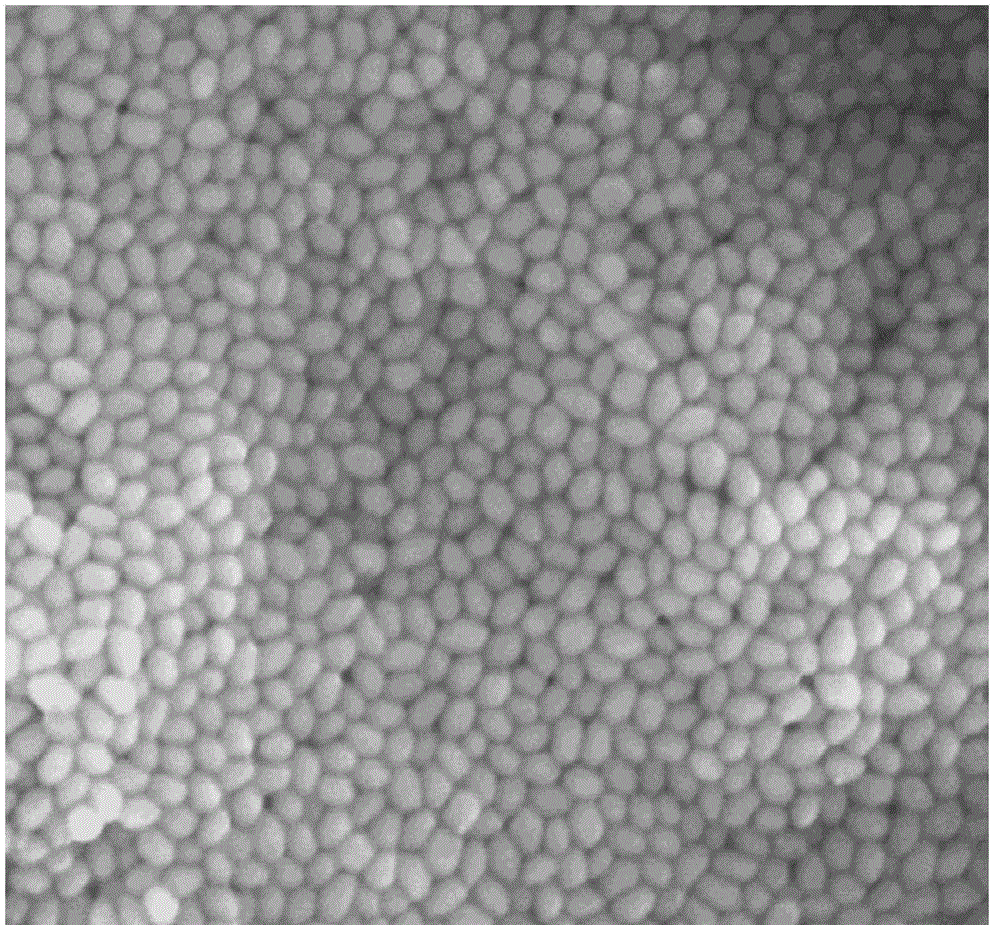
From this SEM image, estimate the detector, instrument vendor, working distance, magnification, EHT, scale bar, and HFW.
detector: TLD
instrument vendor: FEI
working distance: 5.2 mm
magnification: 100 K X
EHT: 10 kV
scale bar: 500 nm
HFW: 2.4 µm